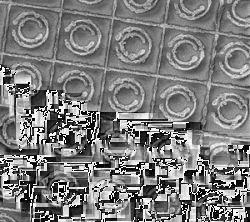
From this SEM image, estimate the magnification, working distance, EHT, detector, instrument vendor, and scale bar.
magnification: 0.95 K X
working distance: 14 mm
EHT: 5 kV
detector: SEI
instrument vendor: JEOL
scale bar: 10000 nm